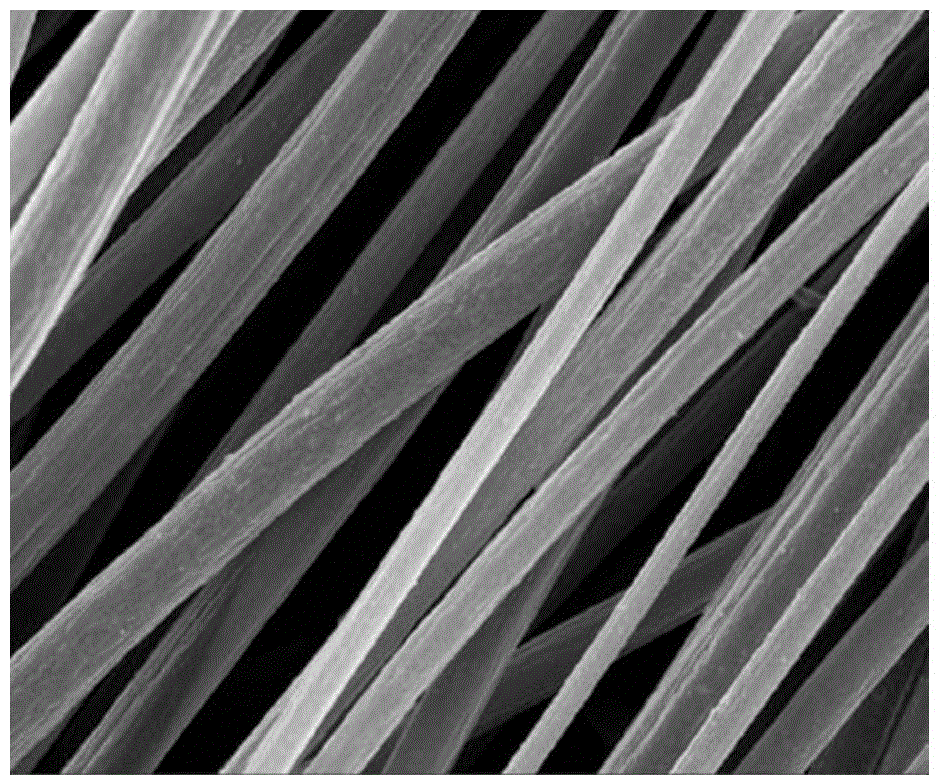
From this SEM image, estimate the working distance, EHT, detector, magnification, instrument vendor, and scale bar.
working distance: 7 mm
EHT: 10 kV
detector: InLens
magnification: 5 K X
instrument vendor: Zeiss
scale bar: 1000 nm